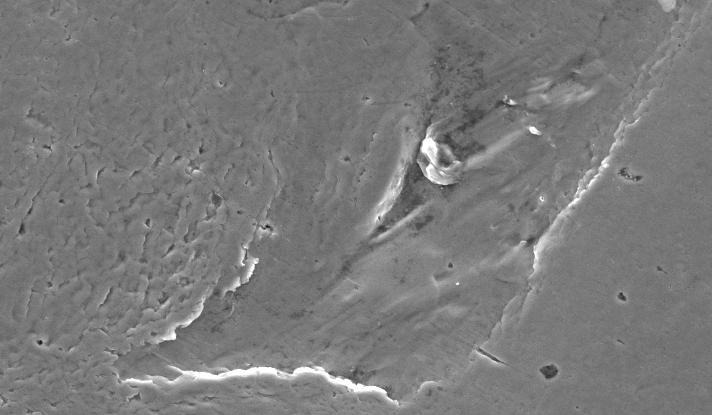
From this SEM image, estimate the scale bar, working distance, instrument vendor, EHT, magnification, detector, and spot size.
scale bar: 20000 nm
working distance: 12.7 mm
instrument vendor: FEI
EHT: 20 kV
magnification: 1 K X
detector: SE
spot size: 3.9